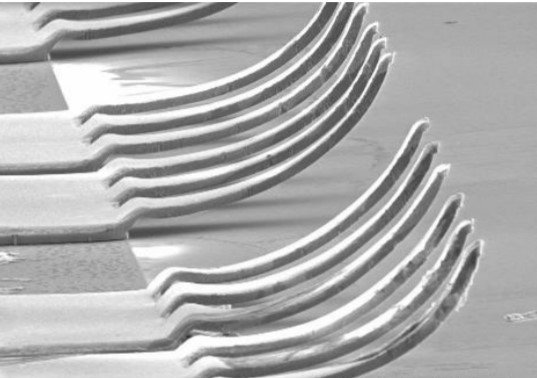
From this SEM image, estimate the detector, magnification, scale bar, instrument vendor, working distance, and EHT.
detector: SE(U)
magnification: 0.4 K X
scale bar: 100000 nm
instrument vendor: Hitachi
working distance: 14.4 mm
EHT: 10 kV